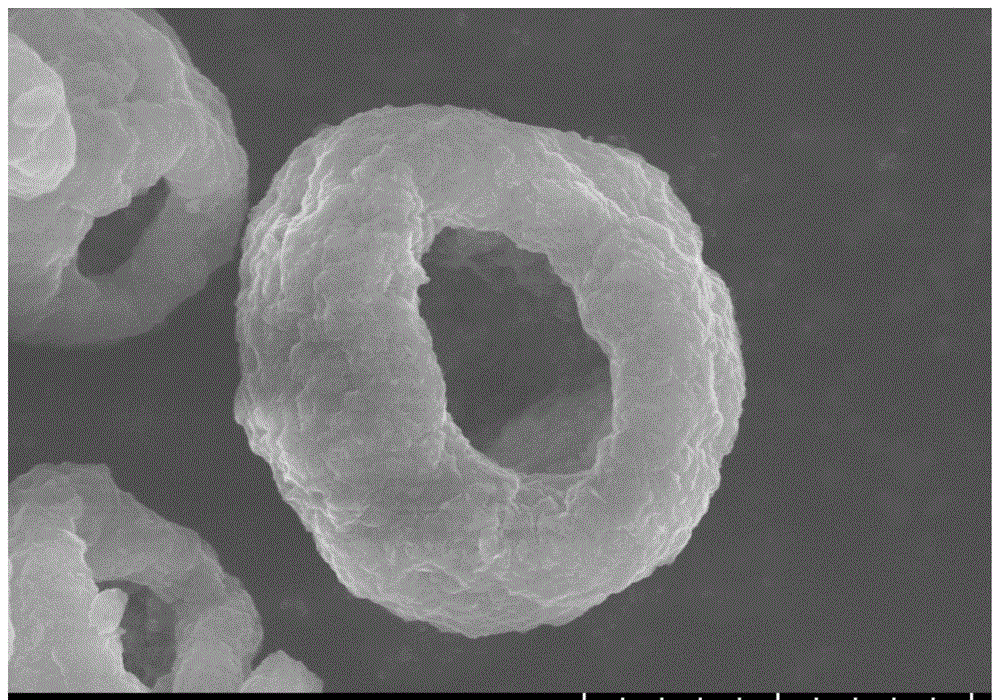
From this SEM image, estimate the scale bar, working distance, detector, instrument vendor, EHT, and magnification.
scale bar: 5000 nm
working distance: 8.8 mm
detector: SE(UL)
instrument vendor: Hitachi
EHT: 10 kV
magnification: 10 K X